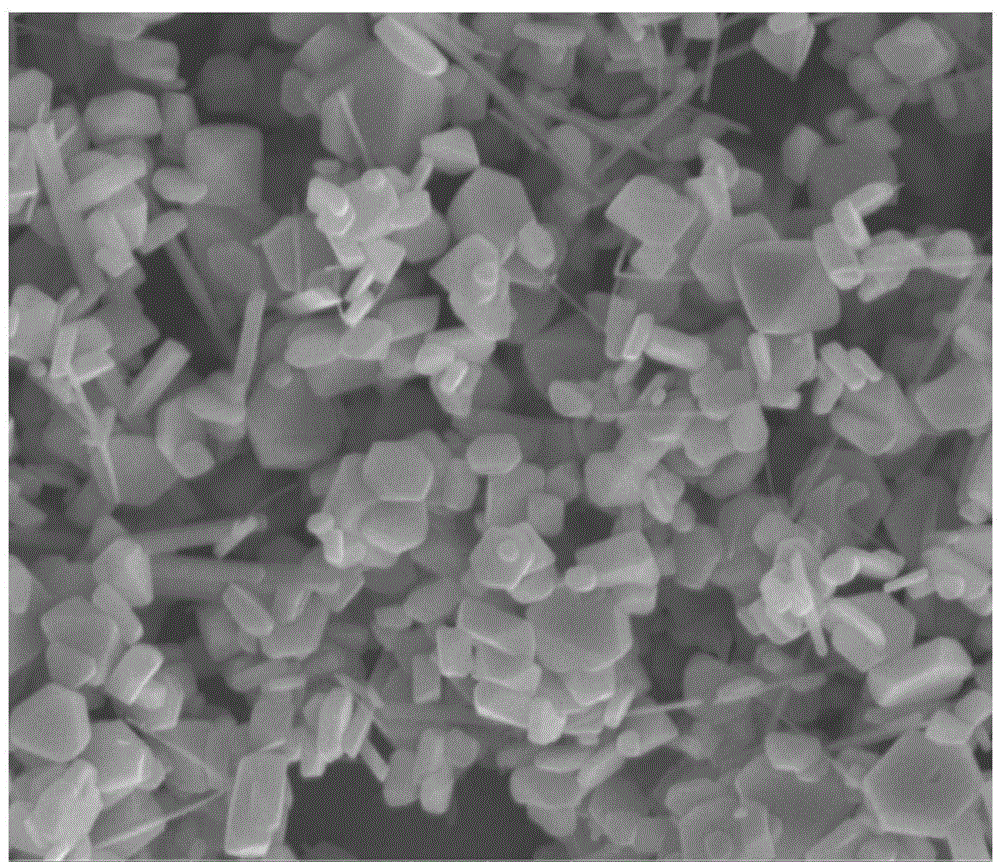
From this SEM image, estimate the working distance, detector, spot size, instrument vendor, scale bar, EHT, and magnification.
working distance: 5.3 mm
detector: ETD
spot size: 2.5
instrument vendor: FEI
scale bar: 2000 nm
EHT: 10 kV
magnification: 40 K X